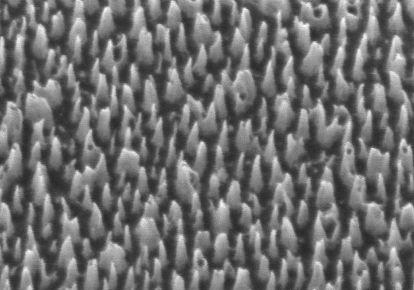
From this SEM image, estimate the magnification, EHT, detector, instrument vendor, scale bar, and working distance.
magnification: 40 K X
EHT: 1 kV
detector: SE(U)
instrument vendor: Hitachi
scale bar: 1000 nm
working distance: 8.6 mm